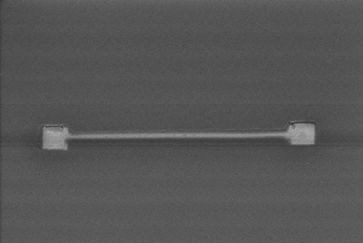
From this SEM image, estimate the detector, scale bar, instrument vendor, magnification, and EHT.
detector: SE(M)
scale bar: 2000 nm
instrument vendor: Hitachi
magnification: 20 K X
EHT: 10 kV